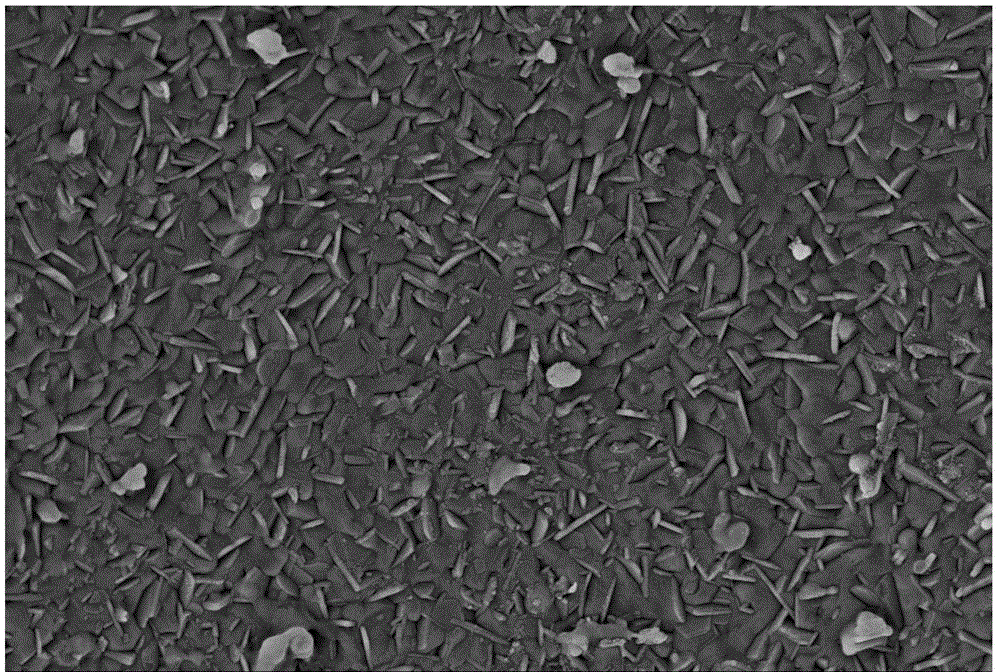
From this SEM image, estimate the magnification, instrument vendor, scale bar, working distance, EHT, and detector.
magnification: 10 K X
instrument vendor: Zeiss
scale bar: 1000 nm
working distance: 7.2 mm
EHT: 5 kV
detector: SE2